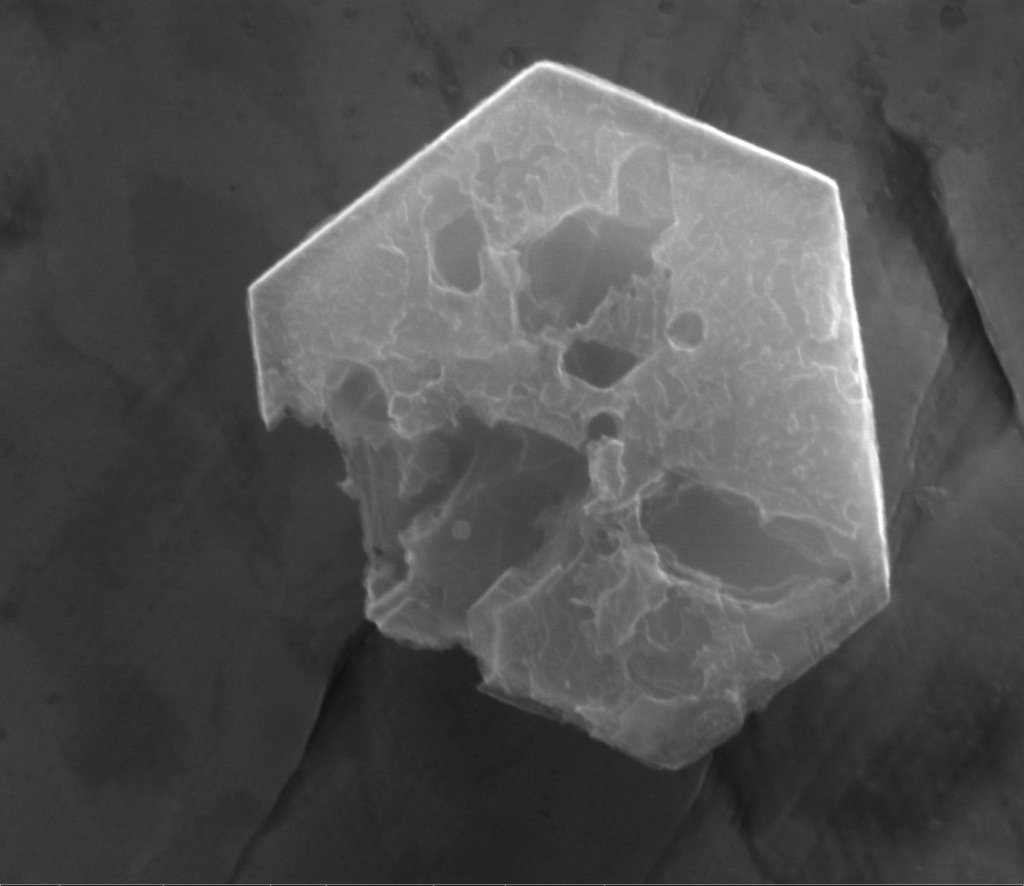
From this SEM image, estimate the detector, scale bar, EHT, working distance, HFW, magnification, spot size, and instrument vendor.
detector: ETD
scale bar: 1000 nm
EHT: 30 kV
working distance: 10.9 mm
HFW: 3.4 µm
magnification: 75.382 K X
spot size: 5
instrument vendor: FEI